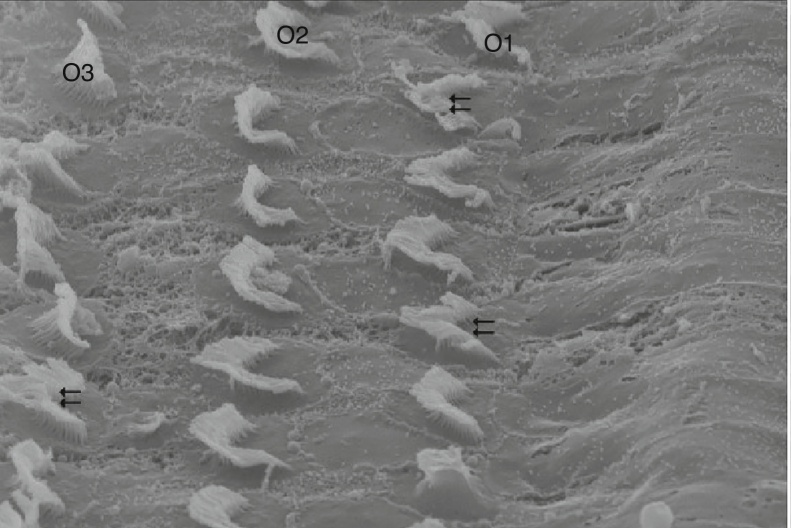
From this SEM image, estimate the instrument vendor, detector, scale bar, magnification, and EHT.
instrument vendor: Zeiss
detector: SE1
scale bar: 2000 nm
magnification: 10 K X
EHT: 20 kV